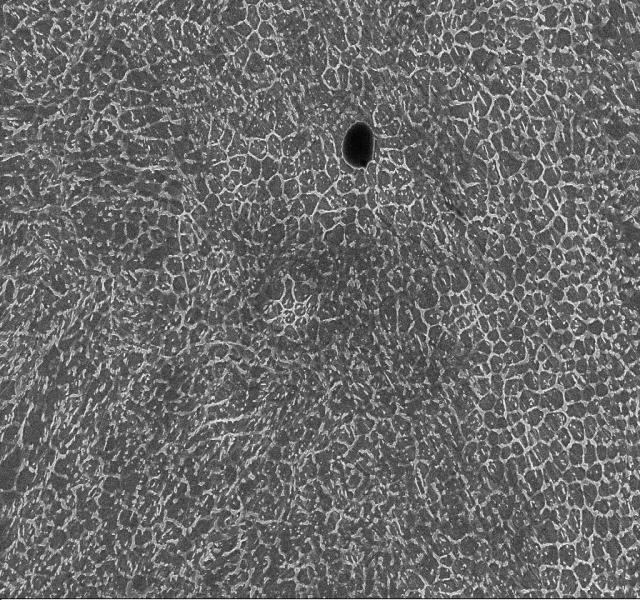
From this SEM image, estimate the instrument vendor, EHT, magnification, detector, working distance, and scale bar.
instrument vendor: Zeiss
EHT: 15 kV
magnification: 10 K X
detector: InLens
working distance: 7.8 mm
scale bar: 2000 nm